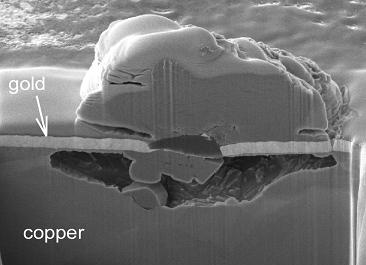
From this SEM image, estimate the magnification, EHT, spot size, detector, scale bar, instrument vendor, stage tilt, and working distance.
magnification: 12 K X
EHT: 5 kV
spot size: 3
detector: CDM-E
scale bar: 2000 nm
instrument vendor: FEI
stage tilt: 52°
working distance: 4.709 mm